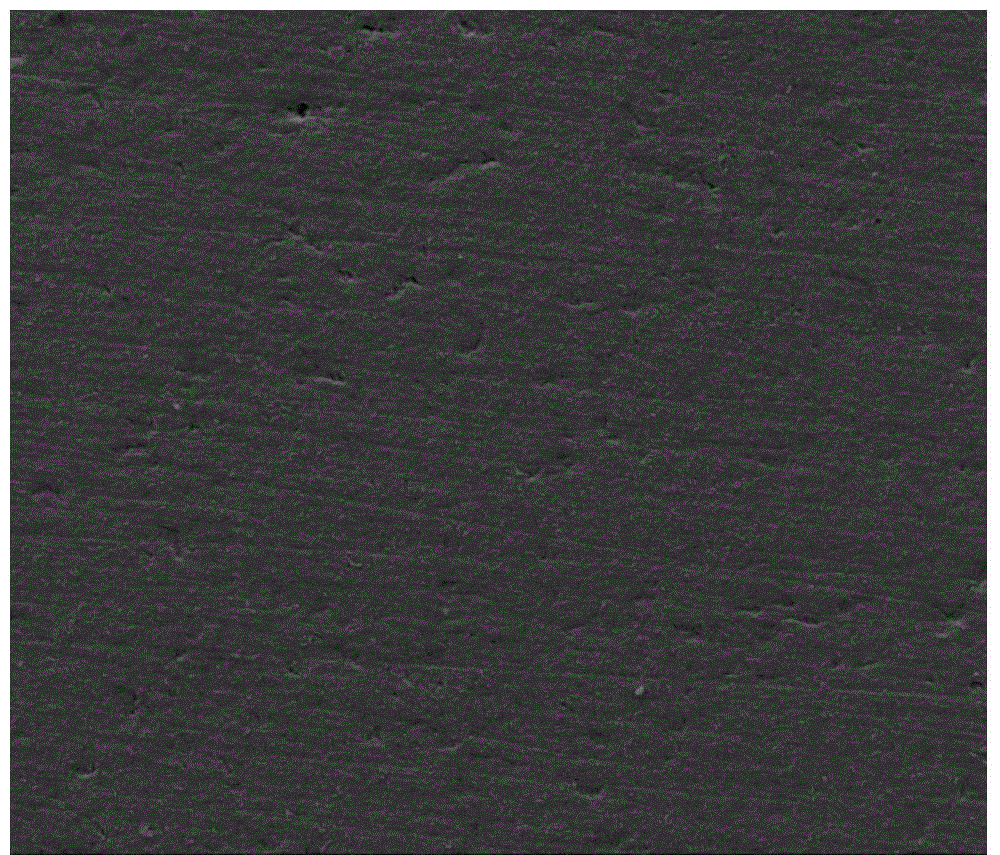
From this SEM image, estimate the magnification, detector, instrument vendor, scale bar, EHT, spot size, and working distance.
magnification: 0.5 K X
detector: ETD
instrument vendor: FEI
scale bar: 200000 nm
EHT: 15 kV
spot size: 4.5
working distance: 12.4 mm